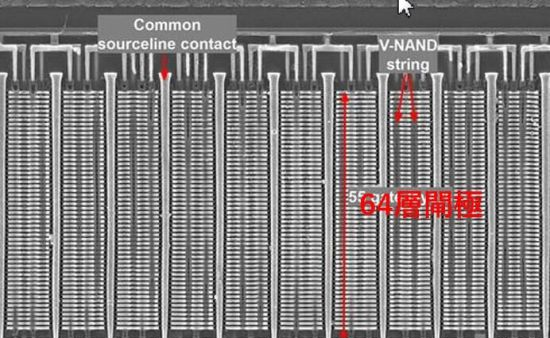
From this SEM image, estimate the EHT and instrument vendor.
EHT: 5 kV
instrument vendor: Zeiss